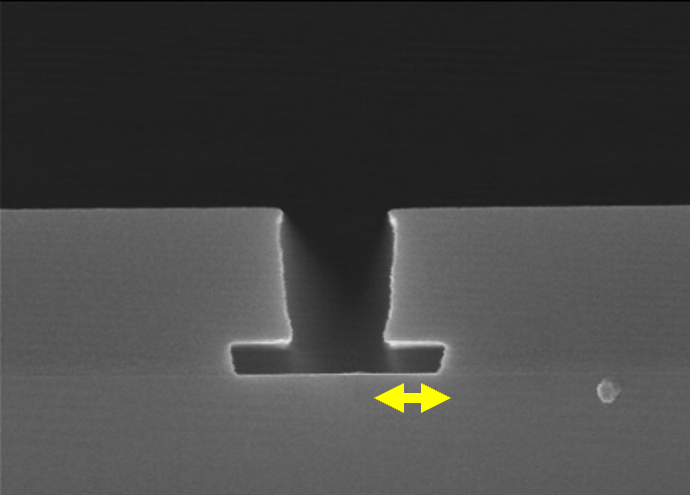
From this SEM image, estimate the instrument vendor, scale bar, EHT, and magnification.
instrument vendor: JEOL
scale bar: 1000 nm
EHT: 15 kV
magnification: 30 K X